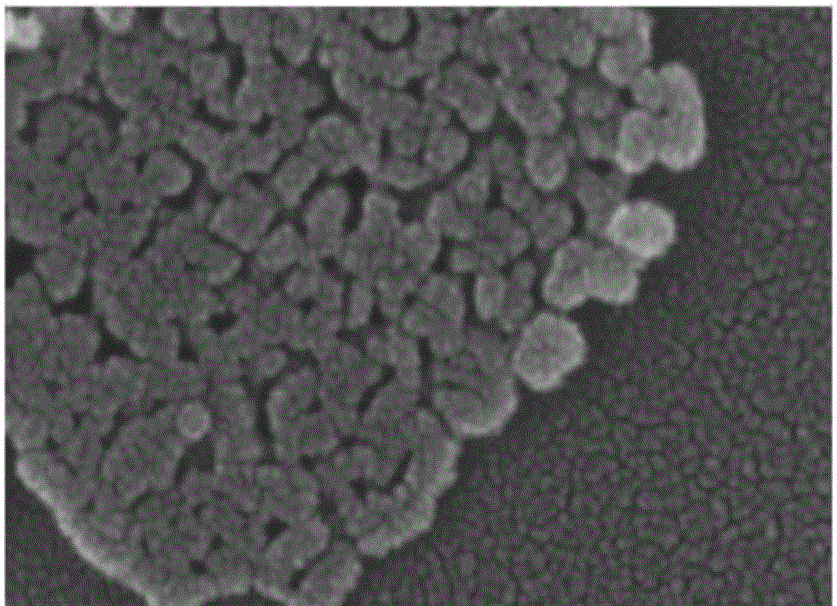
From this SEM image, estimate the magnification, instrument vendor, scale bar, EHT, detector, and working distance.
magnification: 800 K X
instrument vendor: FEI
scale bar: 200 nm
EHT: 15 kV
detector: TLD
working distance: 4.8 mm